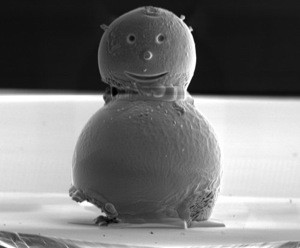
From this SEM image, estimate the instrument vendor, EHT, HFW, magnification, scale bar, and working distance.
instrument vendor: FEI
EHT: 5 kV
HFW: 36.6 µm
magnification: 3.5 K X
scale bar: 10000 nm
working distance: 5.2 mm